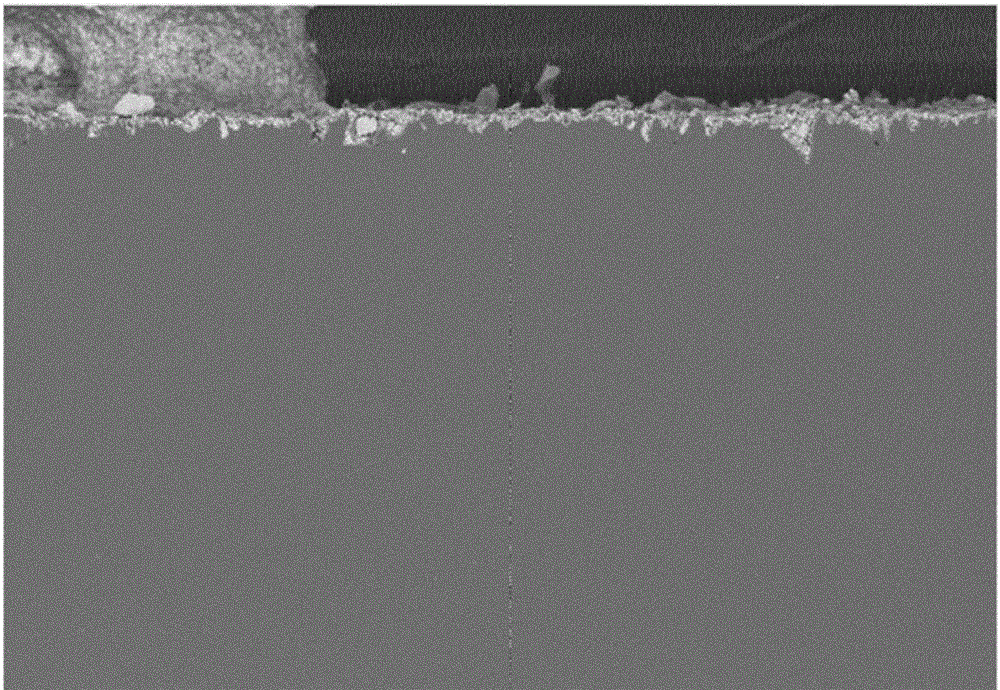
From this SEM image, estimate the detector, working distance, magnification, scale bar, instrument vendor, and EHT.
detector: AsB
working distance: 10 mm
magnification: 0.05 K X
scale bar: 100000 nm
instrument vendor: Zeiss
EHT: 20 kV